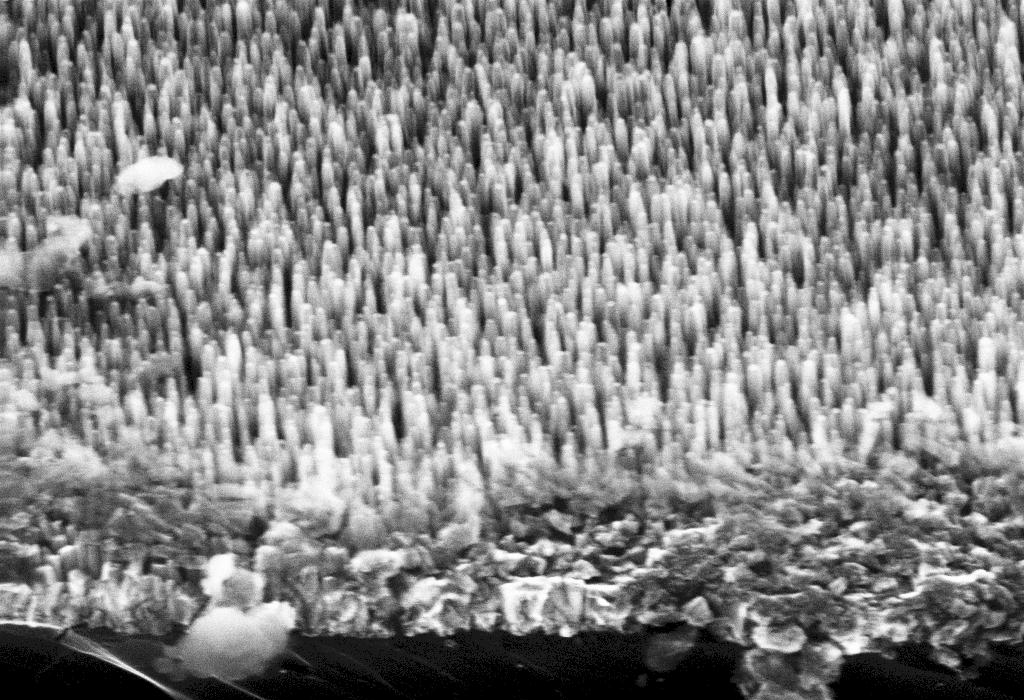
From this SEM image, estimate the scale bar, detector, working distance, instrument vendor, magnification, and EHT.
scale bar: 200 nm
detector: InLens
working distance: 5 mm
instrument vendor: Zeiss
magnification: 100 K X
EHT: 20 kV